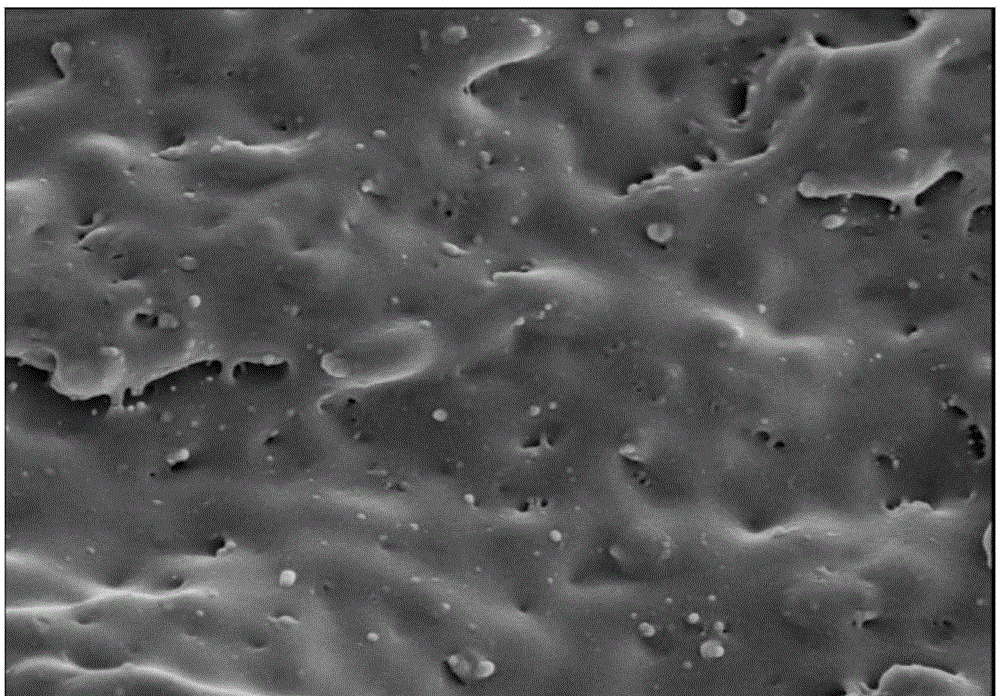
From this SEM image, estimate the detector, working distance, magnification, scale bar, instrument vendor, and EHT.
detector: SE(M)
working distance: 8.8 mm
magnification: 10 K X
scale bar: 5000 nm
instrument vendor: Hitachi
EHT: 5 kV